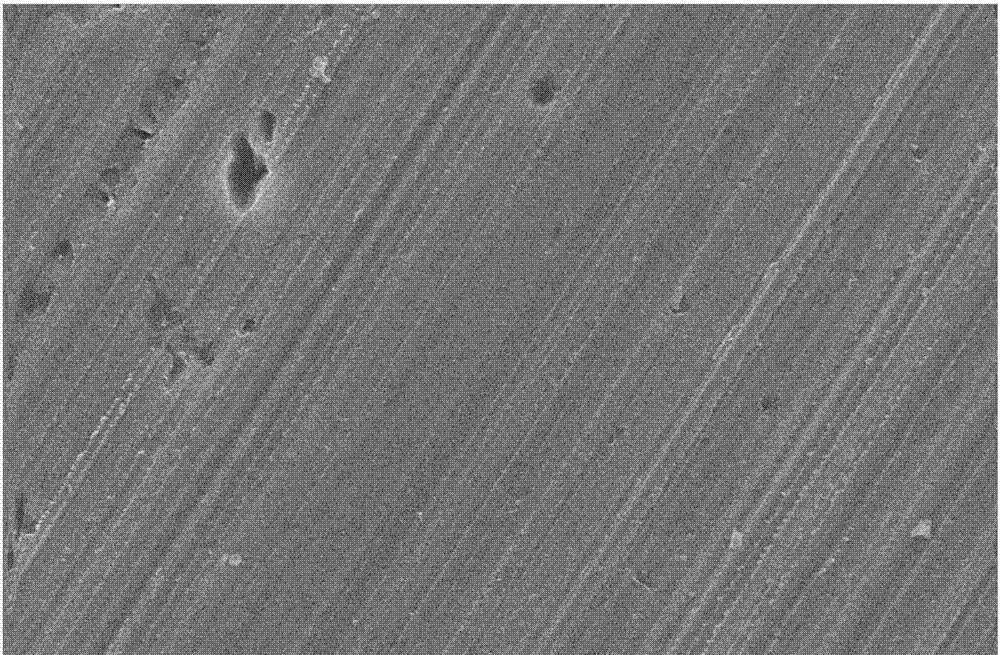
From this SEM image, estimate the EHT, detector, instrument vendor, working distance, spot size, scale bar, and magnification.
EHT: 20 kV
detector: SE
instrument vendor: FEI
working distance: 6.2 mm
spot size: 3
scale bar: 20000 nm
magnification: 1.5 K X